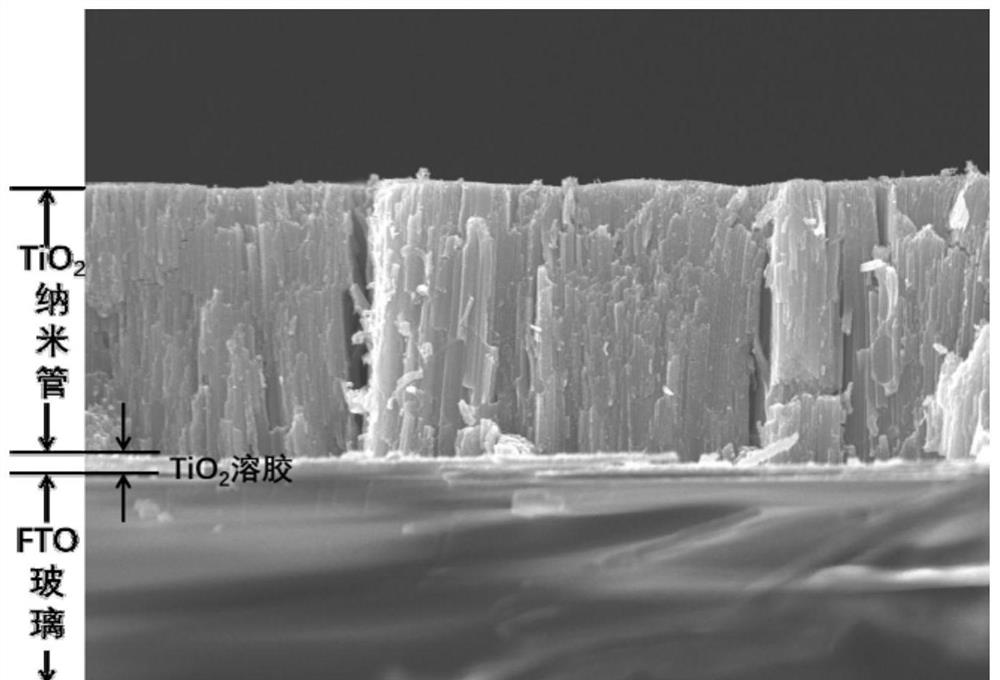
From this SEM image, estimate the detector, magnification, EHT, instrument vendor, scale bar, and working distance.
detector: SEI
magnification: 4.3 K X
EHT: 15 kV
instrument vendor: JEOL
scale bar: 1000 nm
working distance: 9.7 mm